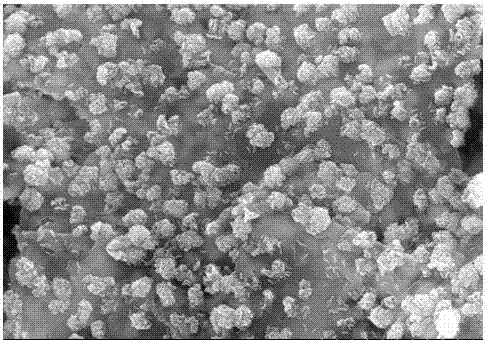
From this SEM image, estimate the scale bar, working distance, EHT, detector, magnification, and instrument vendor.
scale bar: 2000 nm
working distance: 13.1 mm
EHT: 10 kV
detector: SE(M)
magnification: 20 K X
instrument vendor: Hitachi